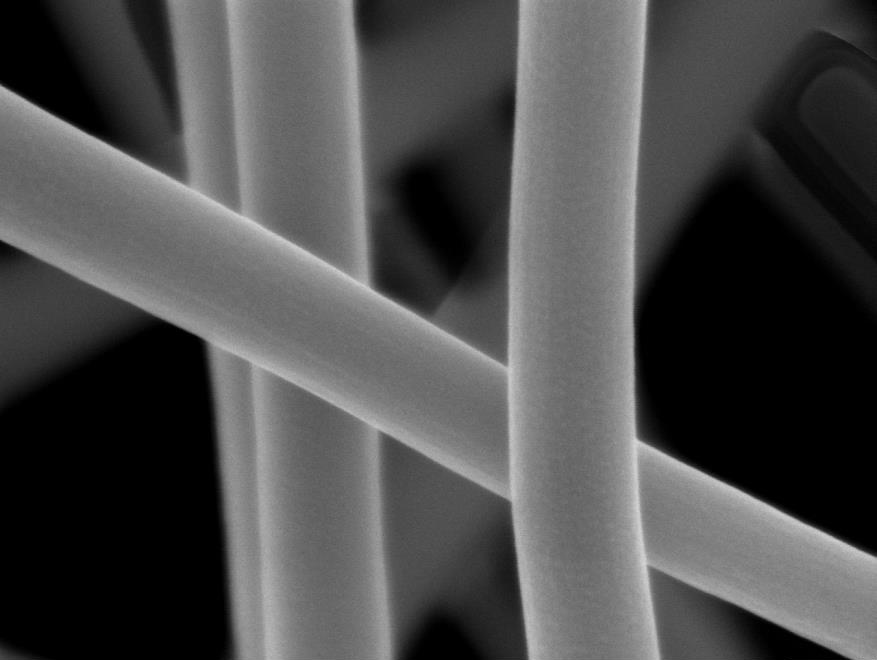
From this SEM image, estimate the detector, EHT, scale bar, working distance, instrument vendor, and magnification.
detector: SEI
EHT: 3 kV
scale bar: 100 nm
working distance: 3 mm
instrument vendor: JEOL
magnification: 100 K X